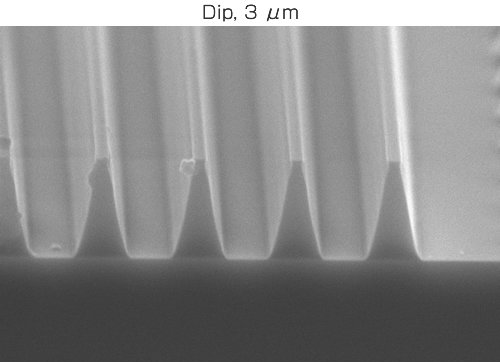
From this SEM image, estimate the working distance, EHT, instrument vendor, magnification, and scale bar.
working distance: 9.5 mm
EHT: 20 kV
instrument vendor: JEOL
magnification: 4 K X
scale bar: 2500 nm